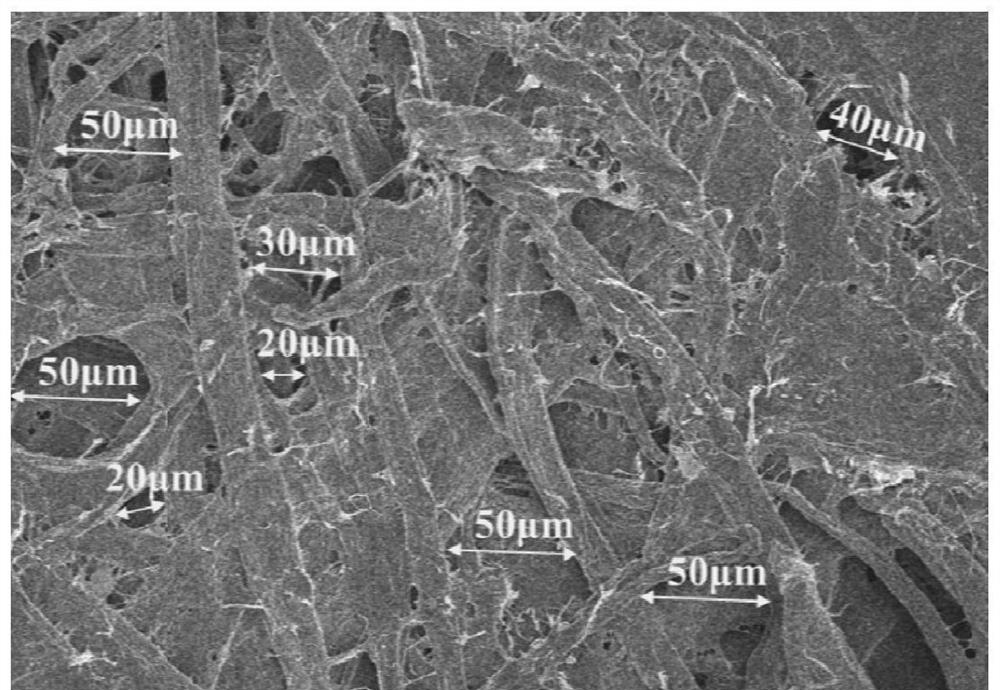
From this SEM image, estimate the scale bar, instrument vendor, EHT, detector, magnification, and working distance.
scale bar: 100000 nm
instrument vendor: Hitachi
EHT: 5 kV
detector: SE(UL)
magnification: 0.4 K X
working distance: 8.7 mm